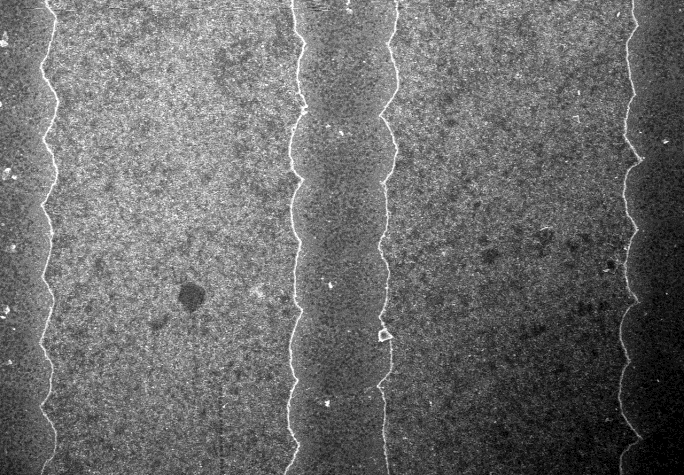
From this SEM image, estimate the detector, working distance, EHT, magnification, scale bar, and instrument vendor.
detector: SE(U,0)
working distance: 12.1 mm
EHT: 20 kV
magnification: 0.25 K X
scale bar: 200000 nm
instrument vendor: Hitachi